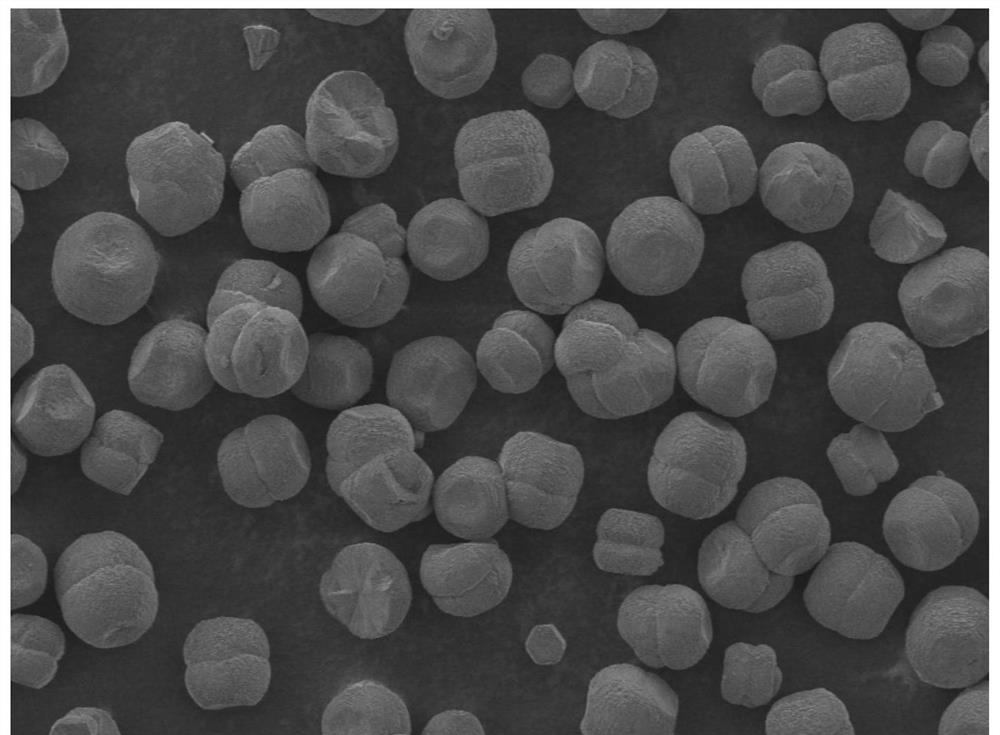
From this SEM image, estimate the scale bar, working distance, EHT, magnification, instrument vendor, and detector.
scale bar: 100000 nm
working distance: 9.5 mm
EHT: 2 kV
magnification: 0.2 K X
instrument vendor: JEOL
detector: LED